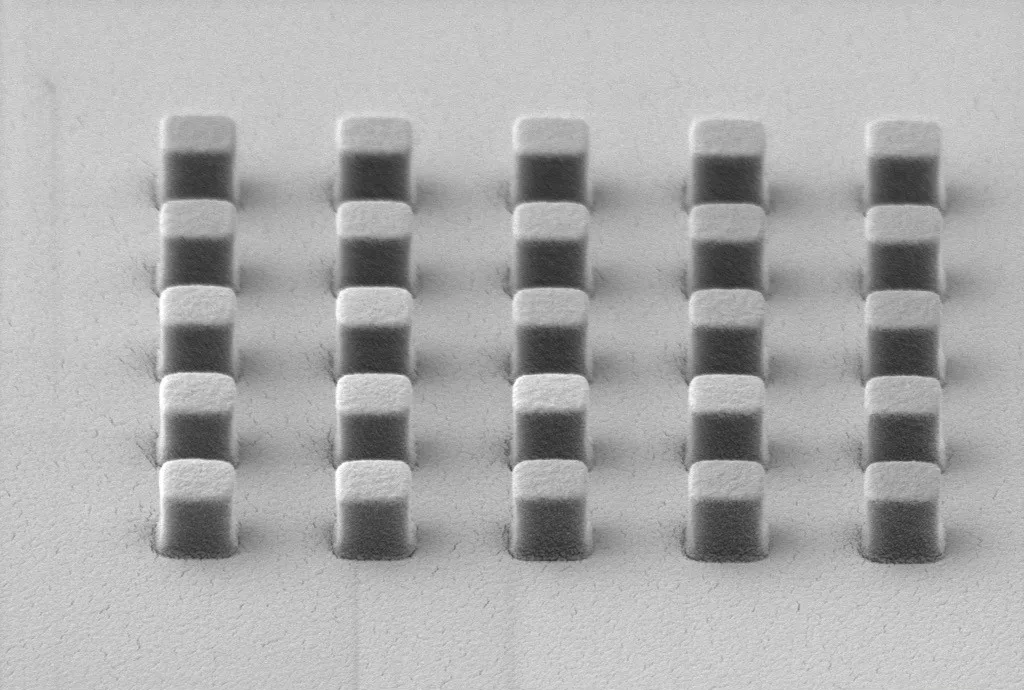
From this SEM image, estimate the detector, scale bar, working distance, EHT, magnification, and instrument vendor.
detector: SE2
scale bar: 1000 nm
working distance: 11.9 mm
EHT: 3 kV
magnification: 30 K X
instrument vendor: Zeiss